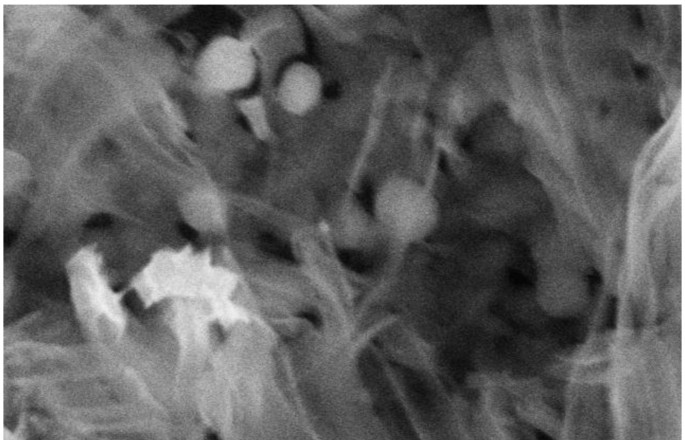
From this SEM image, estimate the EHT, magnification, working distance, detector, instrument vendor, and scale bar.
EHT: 15 kV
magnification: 70 K X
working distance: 13.5 mm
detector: SE(M)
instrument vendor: Hitachi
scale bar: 500 nm